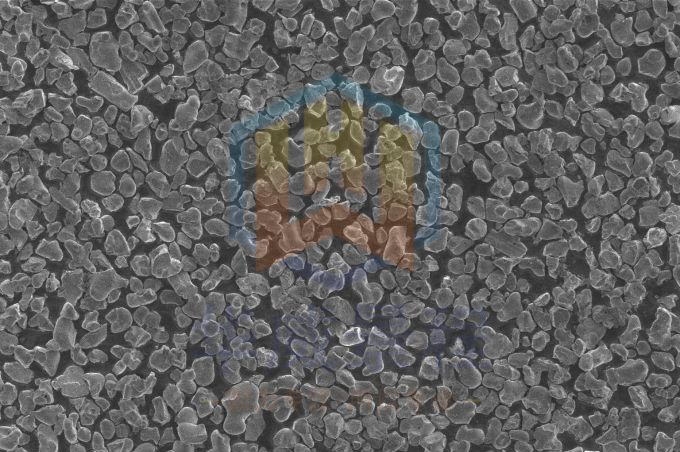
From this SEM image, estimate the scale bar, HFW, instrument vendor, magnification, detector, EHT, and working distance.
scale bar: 30000 nm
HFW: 82.9 µm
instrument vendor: FEI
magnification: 5 K X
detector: ETD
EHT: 2 kV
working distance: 4.3 mm